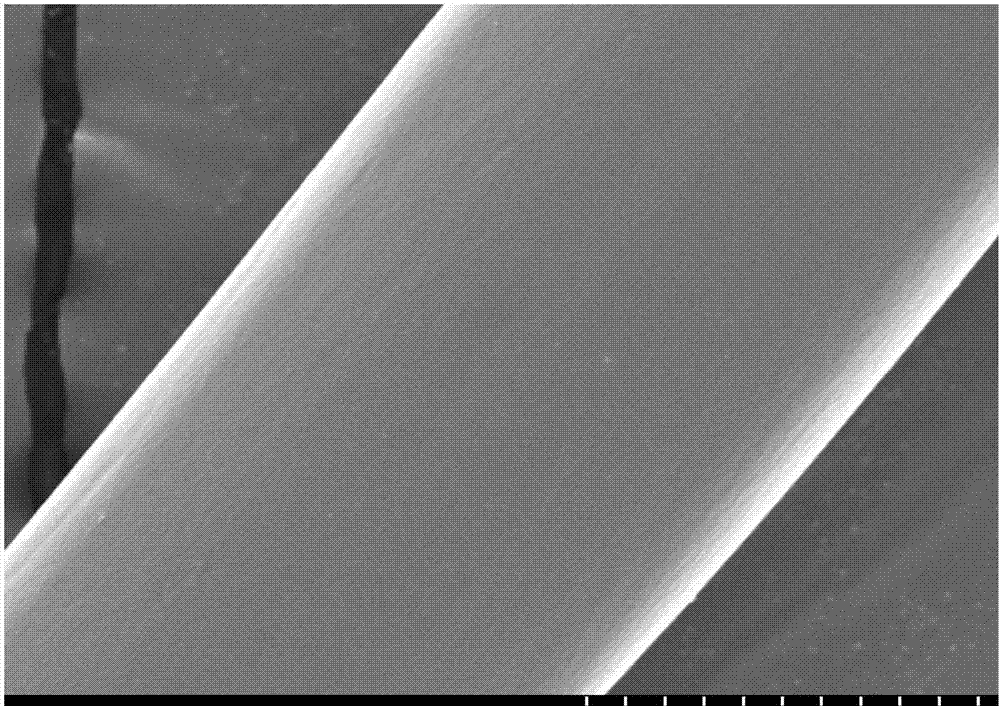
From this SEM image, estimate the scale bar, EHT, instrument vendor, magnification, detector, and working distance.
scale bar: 10000 nm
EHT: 5 kV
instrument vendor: Hitachi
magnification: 5 K X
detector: SE(U)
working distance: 9.1 mm